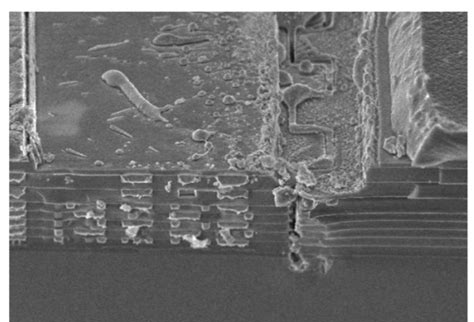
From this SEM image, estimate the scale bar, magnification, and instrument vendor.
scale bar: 7510 nm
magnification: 4 K X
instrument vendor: Hitachi